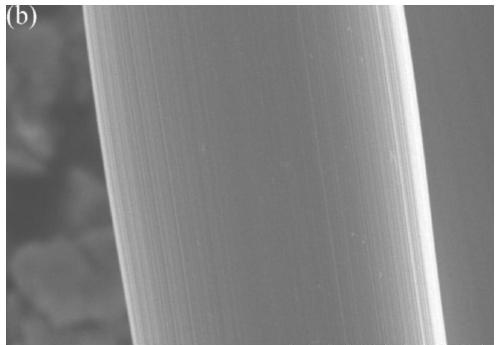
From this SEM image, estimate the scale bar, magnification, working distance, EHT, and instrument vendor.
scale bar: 10000 nm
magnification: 5 K X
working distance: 10.2 mm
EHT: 20 kV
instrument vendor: Hitachi